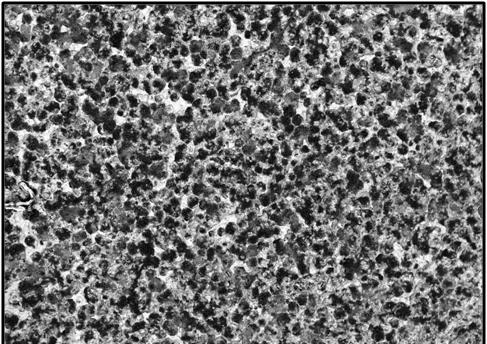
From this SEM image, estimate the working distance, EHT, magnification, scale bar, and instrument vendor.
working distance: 15.9 mm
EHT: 20 kV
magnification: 0.05 K X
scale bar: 1000000 nm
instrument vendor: JEOL